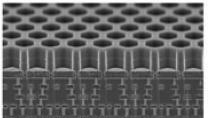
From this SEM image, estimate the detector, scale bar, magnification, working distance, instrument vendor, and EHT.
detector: SE(U)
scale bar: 5000 nm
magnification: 10 K X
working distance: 2.7 mm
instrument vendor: Hitachi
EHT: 5 kV